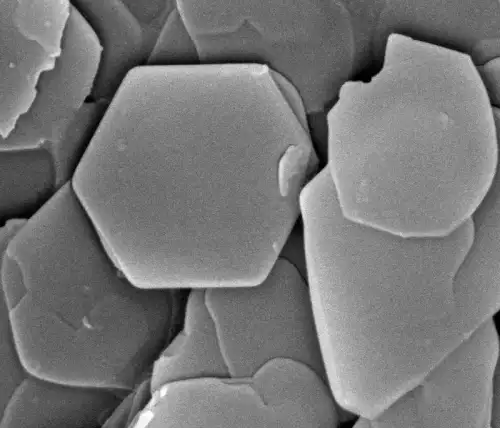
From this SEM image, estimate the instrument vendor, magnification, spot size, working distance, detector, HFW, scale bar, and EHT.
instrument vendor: FEI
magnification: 40 K X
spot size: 2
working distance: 5.7 mm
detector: ETD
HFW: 3.73 µm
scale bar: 1000 nm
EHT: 10 kV